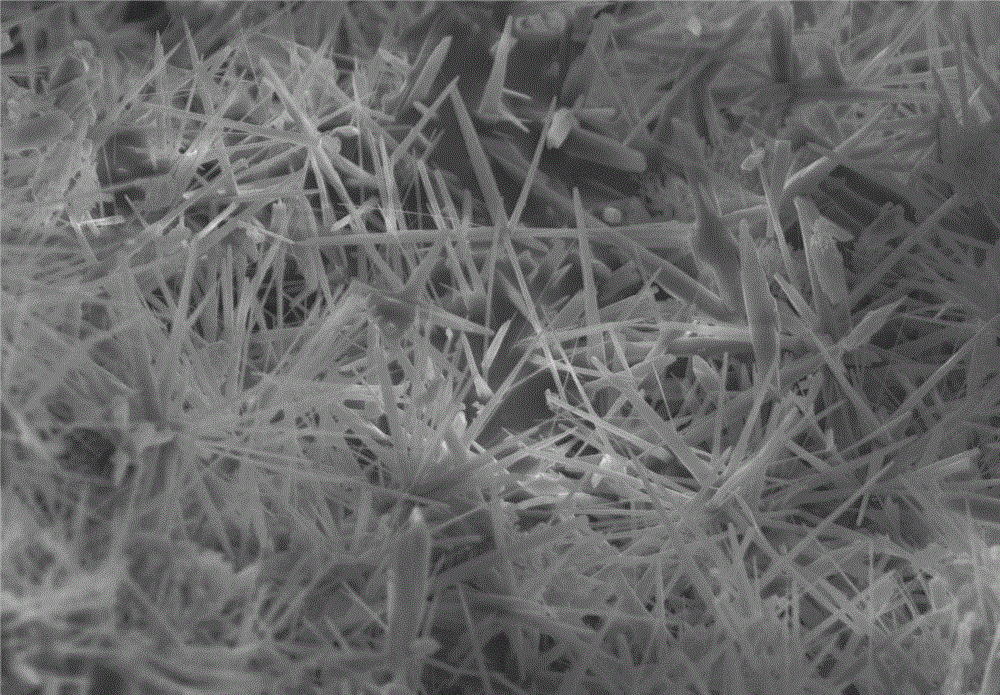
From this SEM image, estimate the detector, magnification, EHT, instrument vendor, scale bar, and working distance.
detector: SE(M)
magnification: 1 K X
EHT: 20 kV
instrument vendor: Hitachi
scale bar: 50000 nm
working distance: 13.1 mm